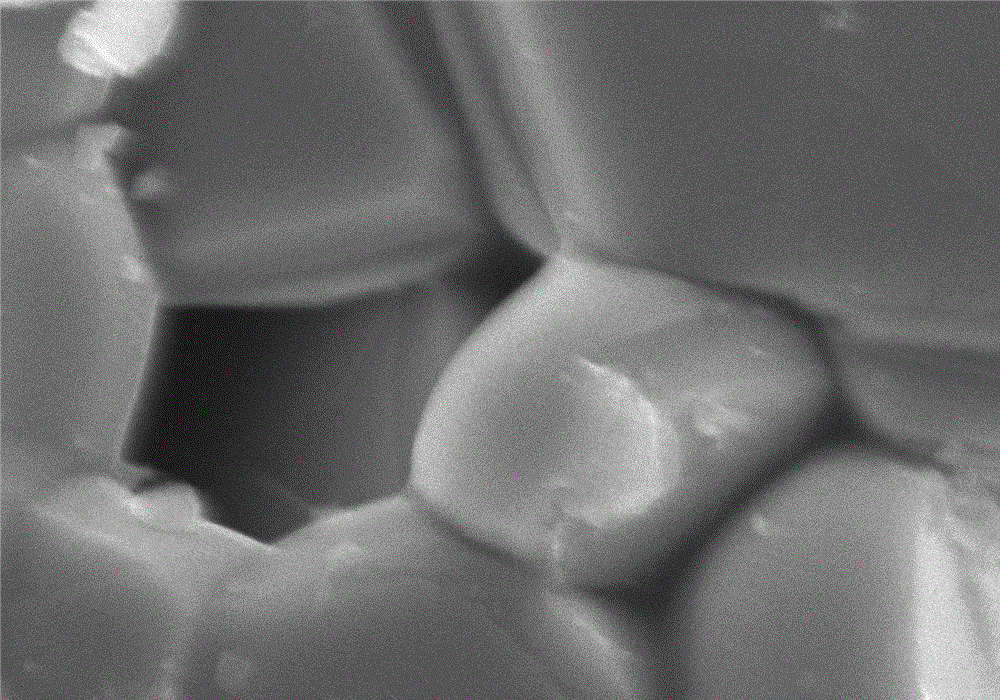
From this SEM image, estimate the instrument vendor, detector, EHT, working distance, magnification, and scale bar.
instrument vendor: Hitachi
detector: SE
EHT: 20 kV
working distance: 10 mm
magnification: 10 K X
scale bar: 5000 nm